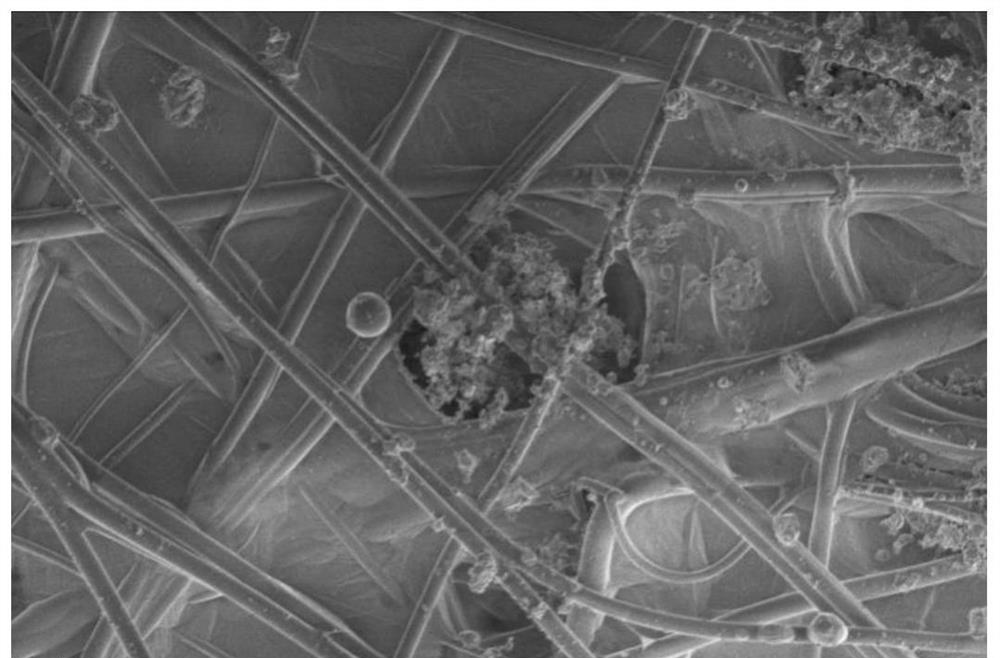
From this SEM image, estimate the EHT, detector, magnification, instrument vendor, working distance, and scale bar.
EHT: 1 kV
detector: ETD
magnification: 2 K X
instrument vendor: FEI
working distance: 5 mm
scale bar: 40000 nm